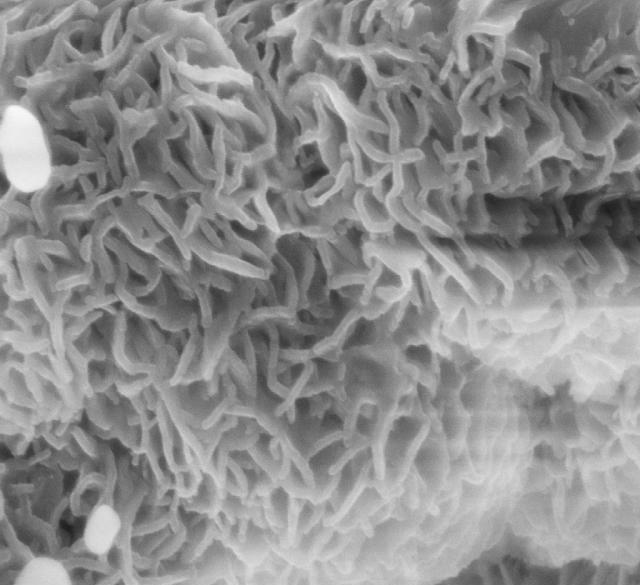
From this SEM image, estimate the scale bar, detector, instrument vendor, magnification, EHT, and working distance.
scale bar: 200 nm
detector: InLens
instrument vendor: Zeiss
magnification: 100 K X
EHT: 5 kV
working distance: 3.3 mm